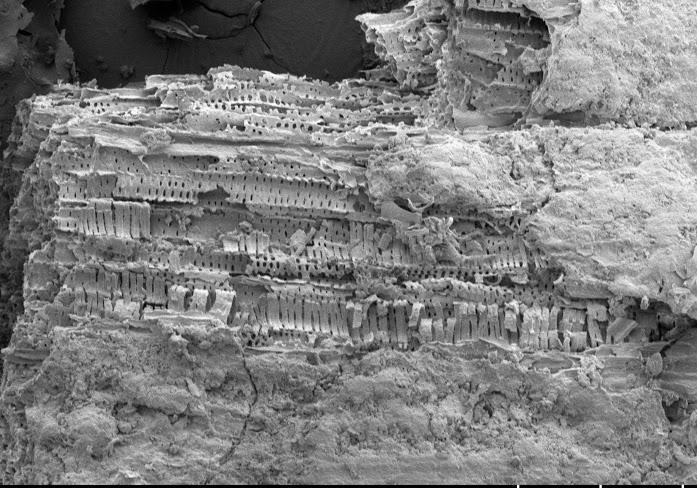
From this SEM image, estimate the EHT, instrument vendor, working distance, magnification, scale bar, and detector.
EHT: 5 kV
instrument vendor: Hitachi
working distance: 8.3 mm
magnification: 0.3 K X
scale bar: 100000 nm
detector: LM(UL)